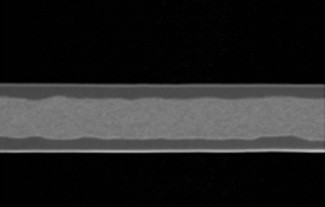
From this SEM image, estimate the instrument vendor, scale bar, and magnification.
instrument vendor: JEOL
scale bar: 5000 nm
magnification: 3 K X